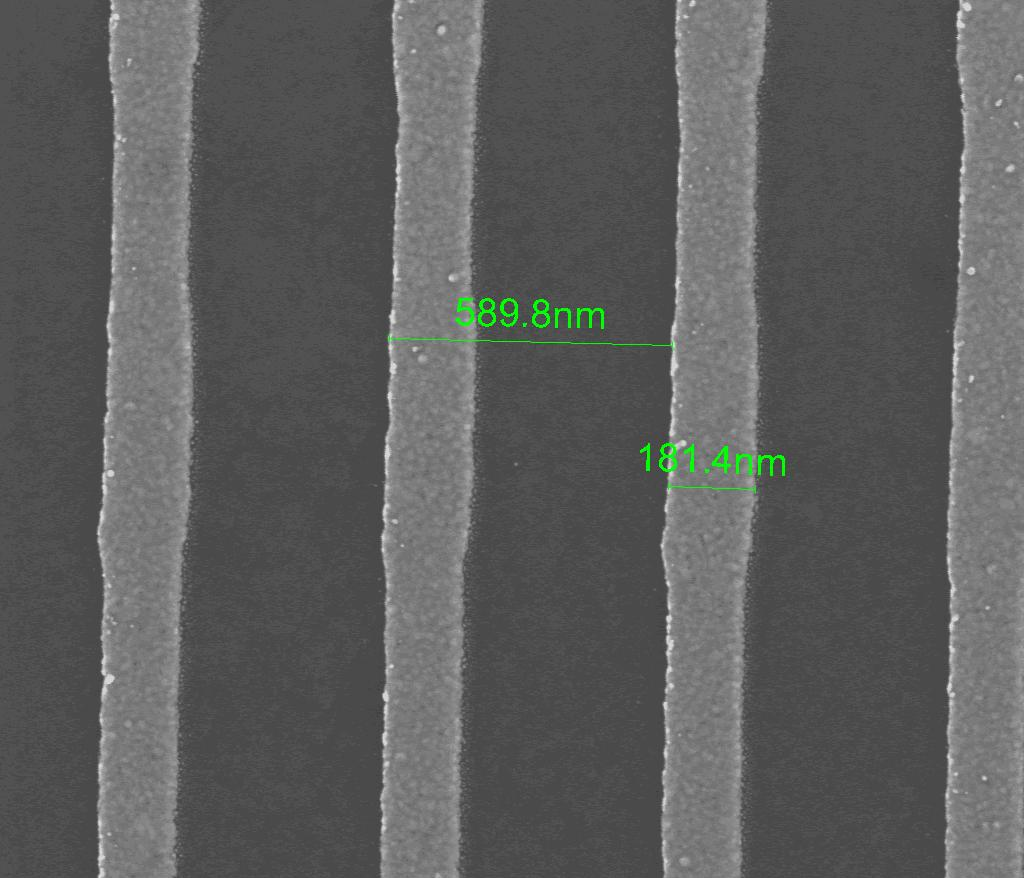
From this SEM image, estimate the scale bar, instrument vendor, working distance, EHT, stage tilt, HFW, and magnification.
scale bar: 500 nm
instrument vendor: FEI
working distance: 5 mm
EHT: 18 kV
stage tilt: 0°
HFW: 2.13 µm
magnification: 120 K X